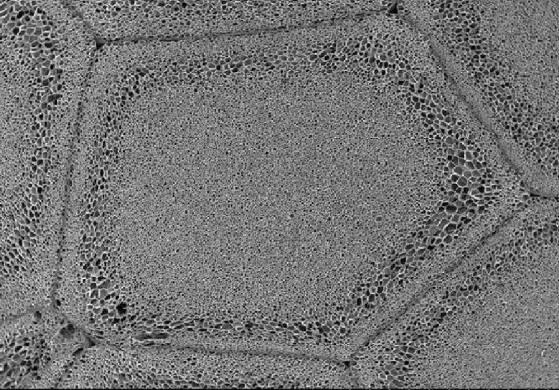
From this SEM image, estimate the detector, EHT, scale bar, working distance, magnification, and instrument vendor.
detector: SE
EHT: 15 kV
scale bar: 3000000 nm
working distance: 34.7 mm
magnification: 0.015 K X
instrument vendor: Hitachi